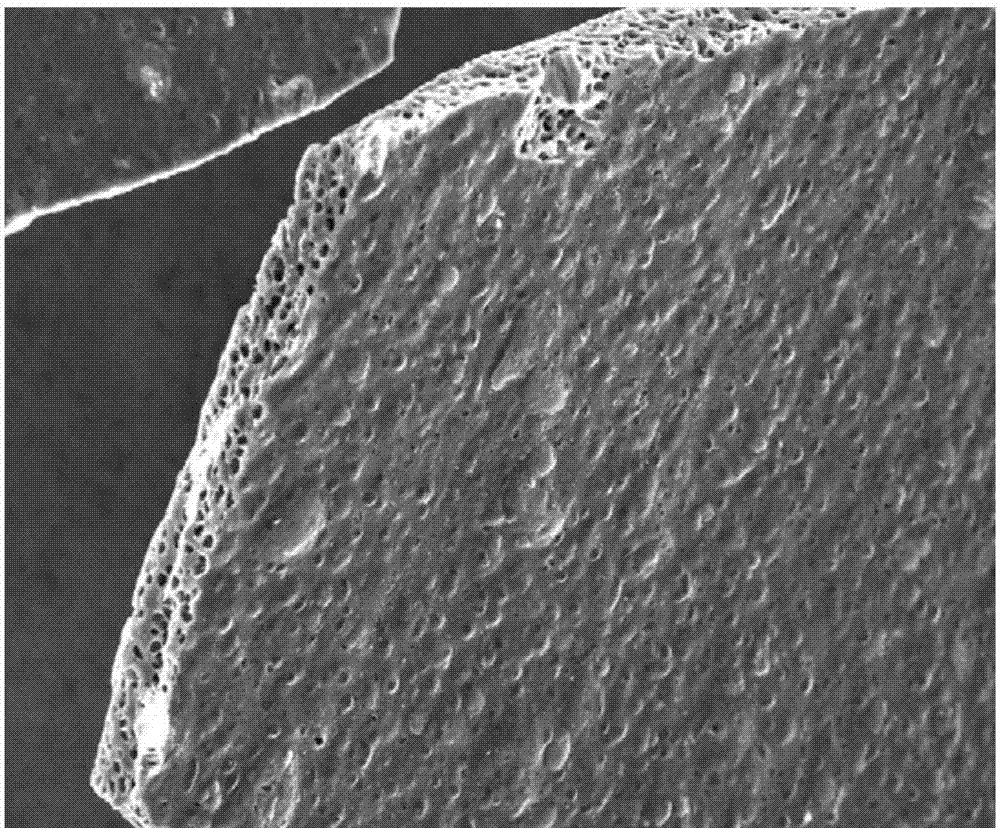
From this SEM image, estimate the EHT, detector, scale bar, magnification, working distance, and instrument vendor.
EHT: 20 kV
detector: InLens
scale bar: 20000 nm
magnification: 0.2 K X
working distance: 5.4 mm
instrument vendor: Zeiss